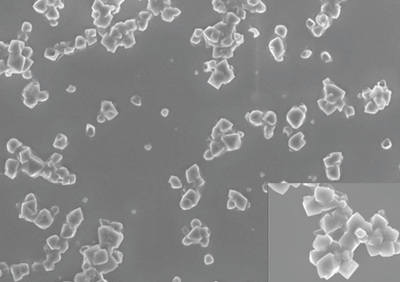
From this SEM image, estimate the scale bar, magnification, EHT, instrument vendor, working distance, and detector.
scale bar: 3000 nm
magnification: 3.8 K X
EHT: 8 kV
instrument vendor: Zeiss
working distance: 8 mm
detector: InLens